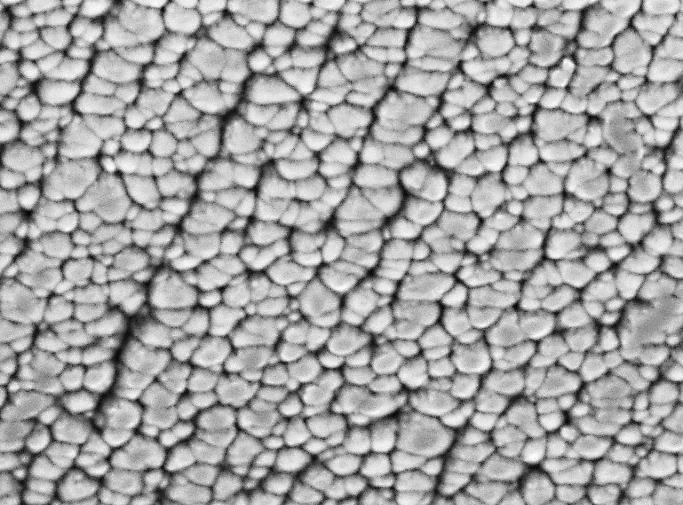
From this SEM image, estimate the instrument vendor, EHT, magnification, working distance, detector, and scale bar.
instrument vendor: JEOL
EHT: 5 kV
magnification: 20 K X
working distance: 10.6 mm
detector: SEI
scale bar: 1000 nm